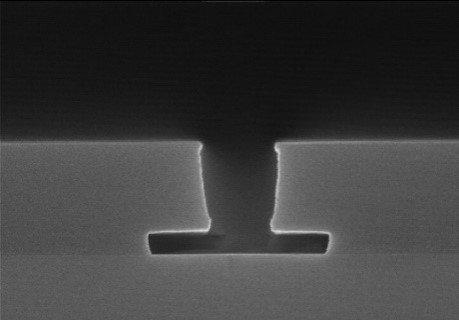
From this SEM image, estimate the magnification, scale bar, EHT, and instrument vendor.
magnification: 30 K X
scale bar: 1000 nm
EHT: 15 kV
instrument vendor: Hitachi